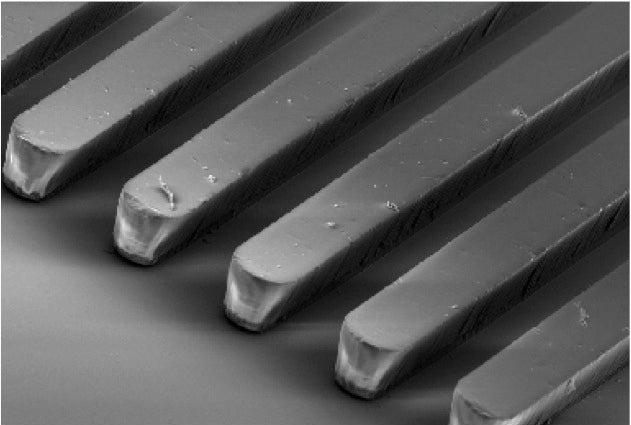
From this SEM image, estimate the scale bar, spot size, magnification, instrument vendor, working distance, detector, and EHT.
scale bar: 100000 nm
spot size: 50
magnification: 0.2 K X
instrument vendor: JEOL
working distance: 32 mm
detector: SEI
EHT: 10 kV